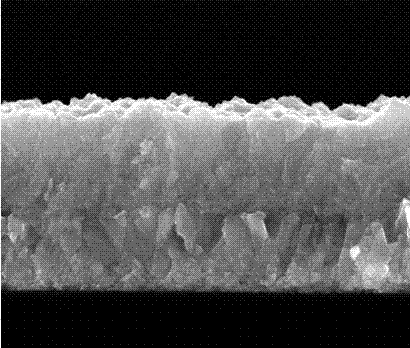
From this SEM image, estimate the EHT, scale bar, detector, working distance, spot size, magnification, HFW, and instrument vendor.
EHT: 15 kV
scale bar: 500 nm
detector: ETD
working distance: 4.8 mm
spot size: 2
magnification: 50 K X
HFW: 2.98 µm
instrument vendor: FEI